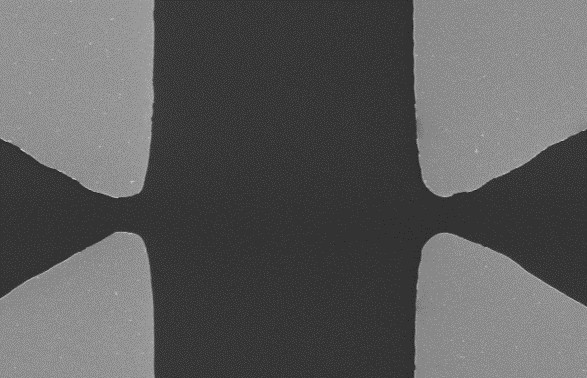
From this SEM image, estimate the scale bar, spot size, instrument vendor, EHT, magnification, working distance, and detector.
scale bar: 20000 nm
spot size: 3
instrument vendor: FEI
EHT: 5 kV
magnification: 1.1 K X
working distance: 6.9 mm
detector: SE